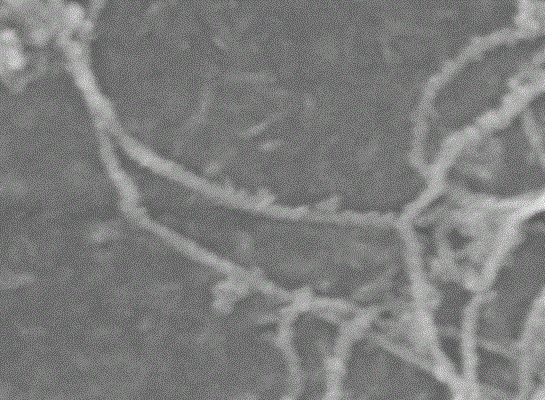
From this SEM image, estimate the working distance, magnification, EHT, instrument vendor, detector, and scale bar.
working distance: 7.8 mm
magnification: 120 K X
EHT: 5 kV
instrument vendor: JEOL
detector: SEI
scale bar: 100 nm